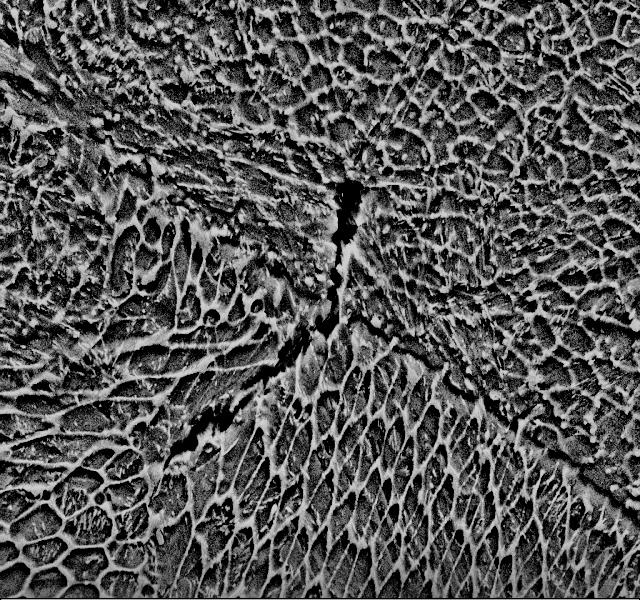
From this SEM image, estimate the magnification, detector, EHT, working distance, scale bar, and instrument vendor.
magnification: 10 K X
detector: SE2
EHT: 15 kV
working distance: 8.3 mm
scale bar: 3000 nm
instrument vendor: Zeiss